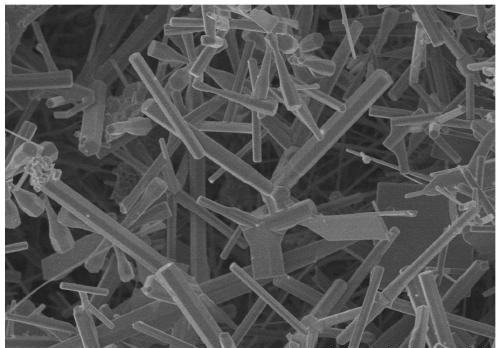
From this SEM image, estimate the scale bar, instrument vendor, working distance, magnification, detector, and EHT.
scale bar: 10000 nm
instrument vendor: Hitachi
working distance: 9.6 mm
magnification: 5 K X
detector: SE(U)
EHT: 5 kV